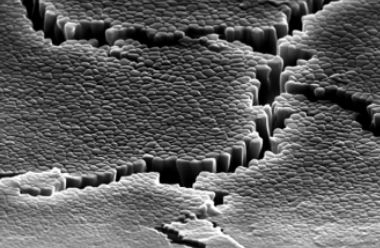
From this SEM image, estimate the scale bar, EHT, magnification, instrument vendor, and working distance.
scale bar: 1000 nm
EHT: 30 kV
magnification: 10 K X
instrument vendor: Zeiss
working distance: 12.4 mm